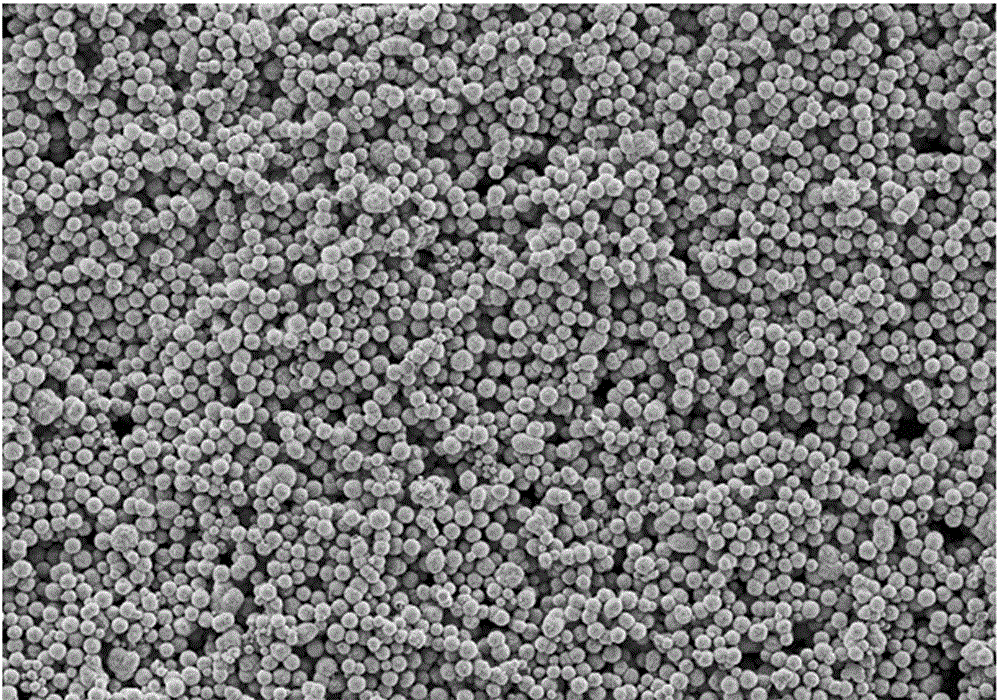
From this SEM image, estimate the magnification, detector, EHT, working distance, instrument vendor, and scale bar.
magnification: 1 K X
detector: SE2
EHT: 3 kV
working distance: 5.5 mm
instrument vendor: Zeiss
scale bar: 10000 nm